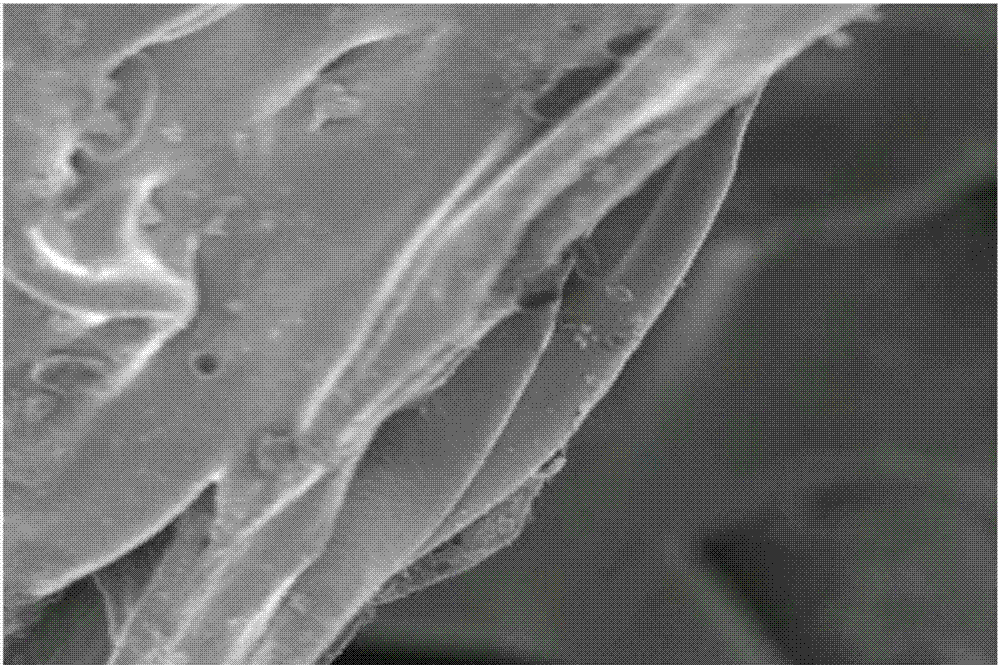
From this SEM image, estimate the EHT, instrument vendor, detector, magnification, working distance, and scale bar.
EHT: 2 kV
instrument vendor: JEOL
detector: SEI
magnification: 1.5 K X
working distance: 6 mm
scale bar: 10000 nm